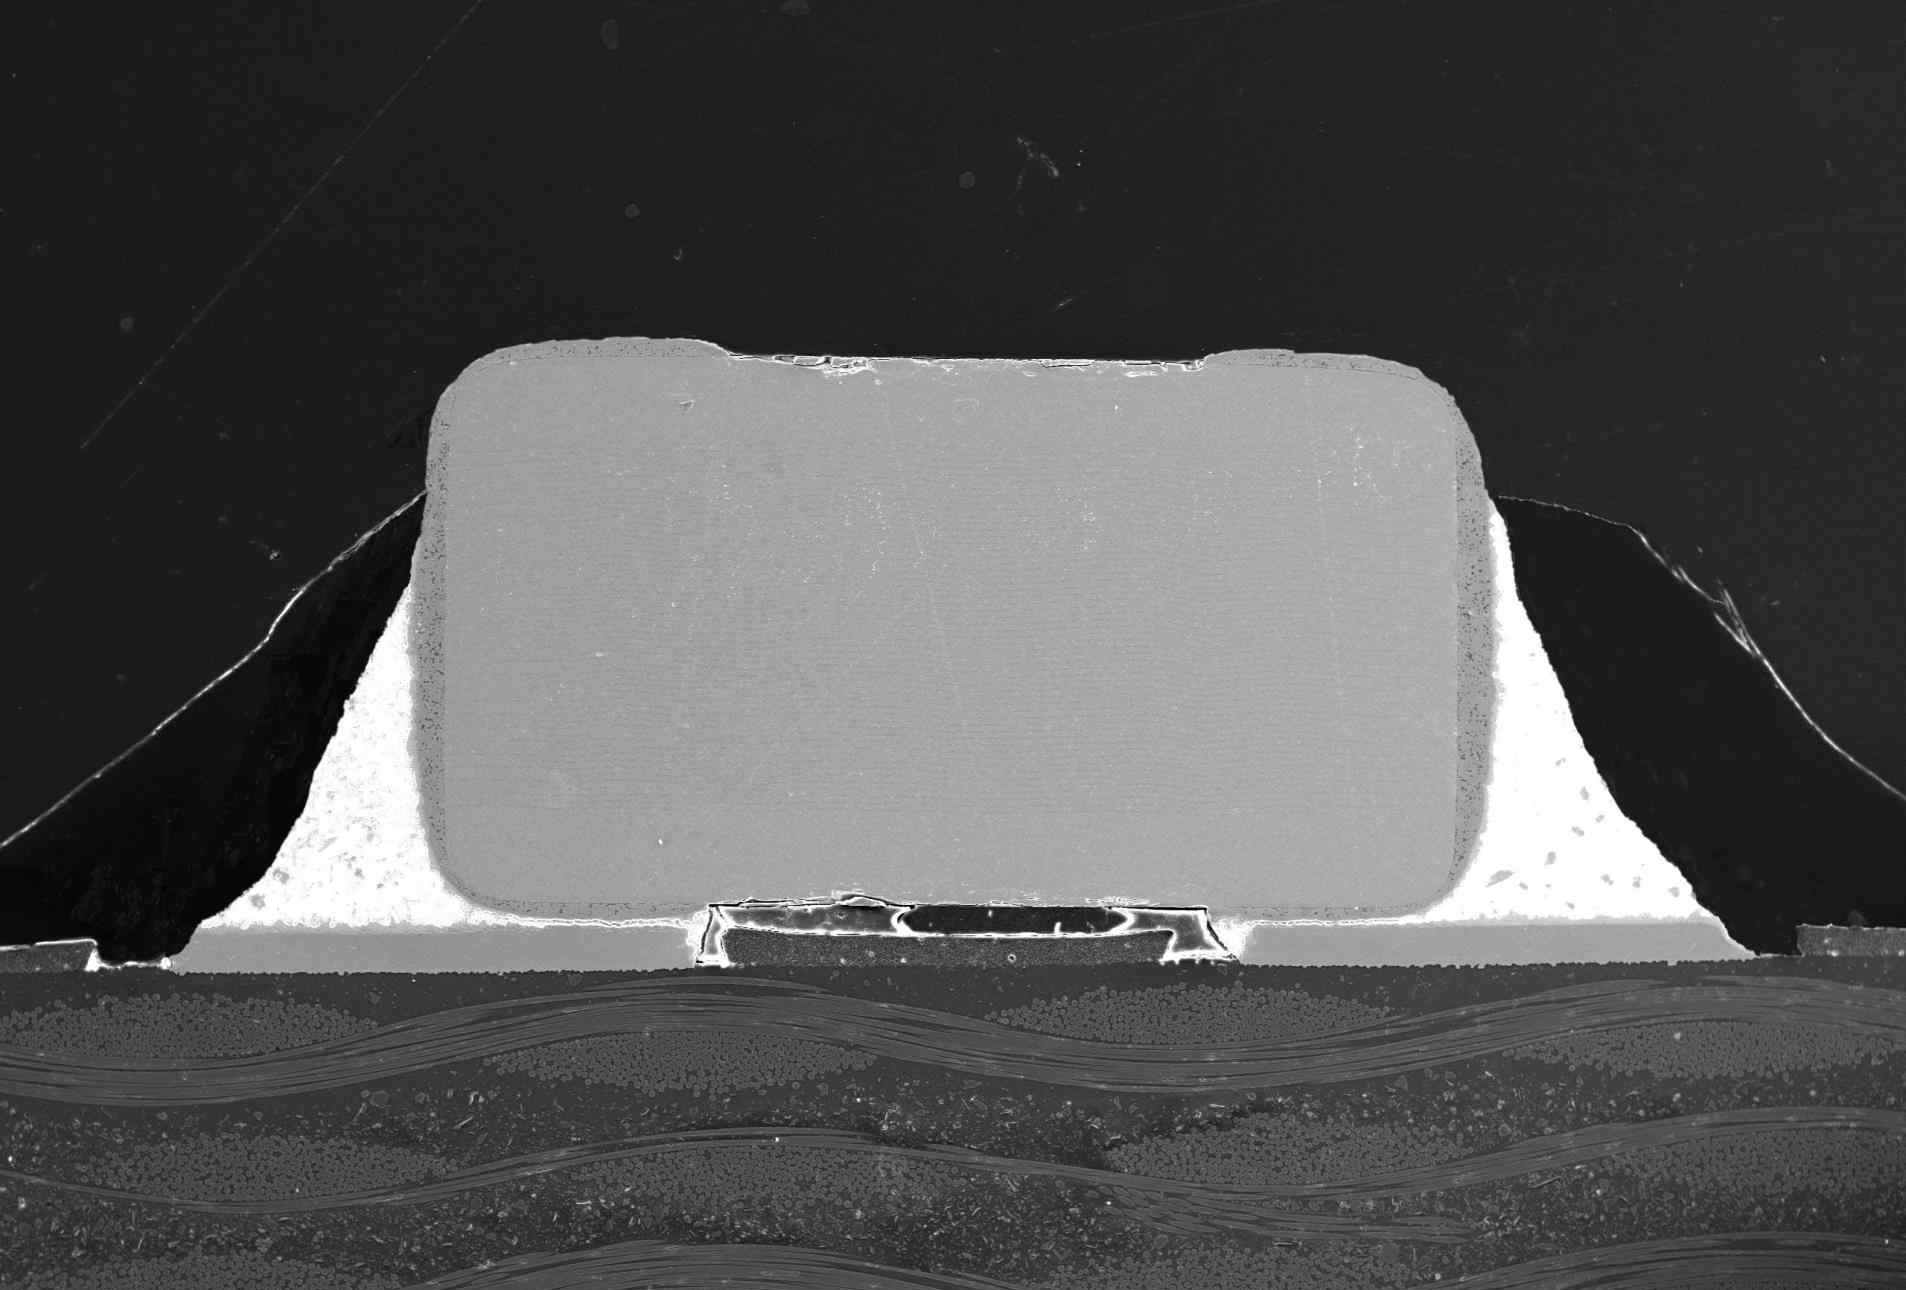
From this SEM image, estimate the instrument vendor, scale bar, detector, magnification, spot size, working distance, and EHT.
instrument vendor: JEOL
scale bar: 500000 nm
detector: SEI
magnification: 0.045 K X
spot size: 70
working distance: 10 mm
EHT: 20 kV